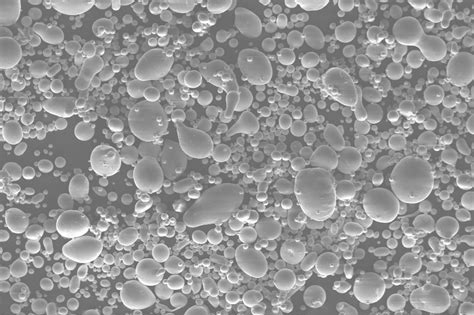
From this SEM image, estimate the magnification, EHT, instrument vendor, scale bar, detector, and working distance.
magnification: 0.2 K X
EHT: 20 kV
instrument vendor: Zeiss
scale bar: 20000 nm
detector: SE1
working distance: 8 mm